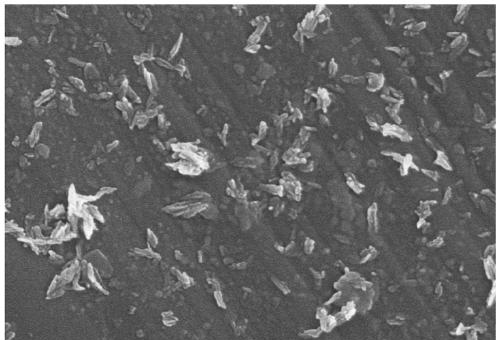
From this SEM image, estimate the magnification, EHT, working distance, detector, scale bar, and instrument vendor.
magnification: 50 K X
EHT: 5 kV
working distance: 7.9 mm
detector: SE(M)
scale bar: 1000 nm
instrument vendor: Hitachi